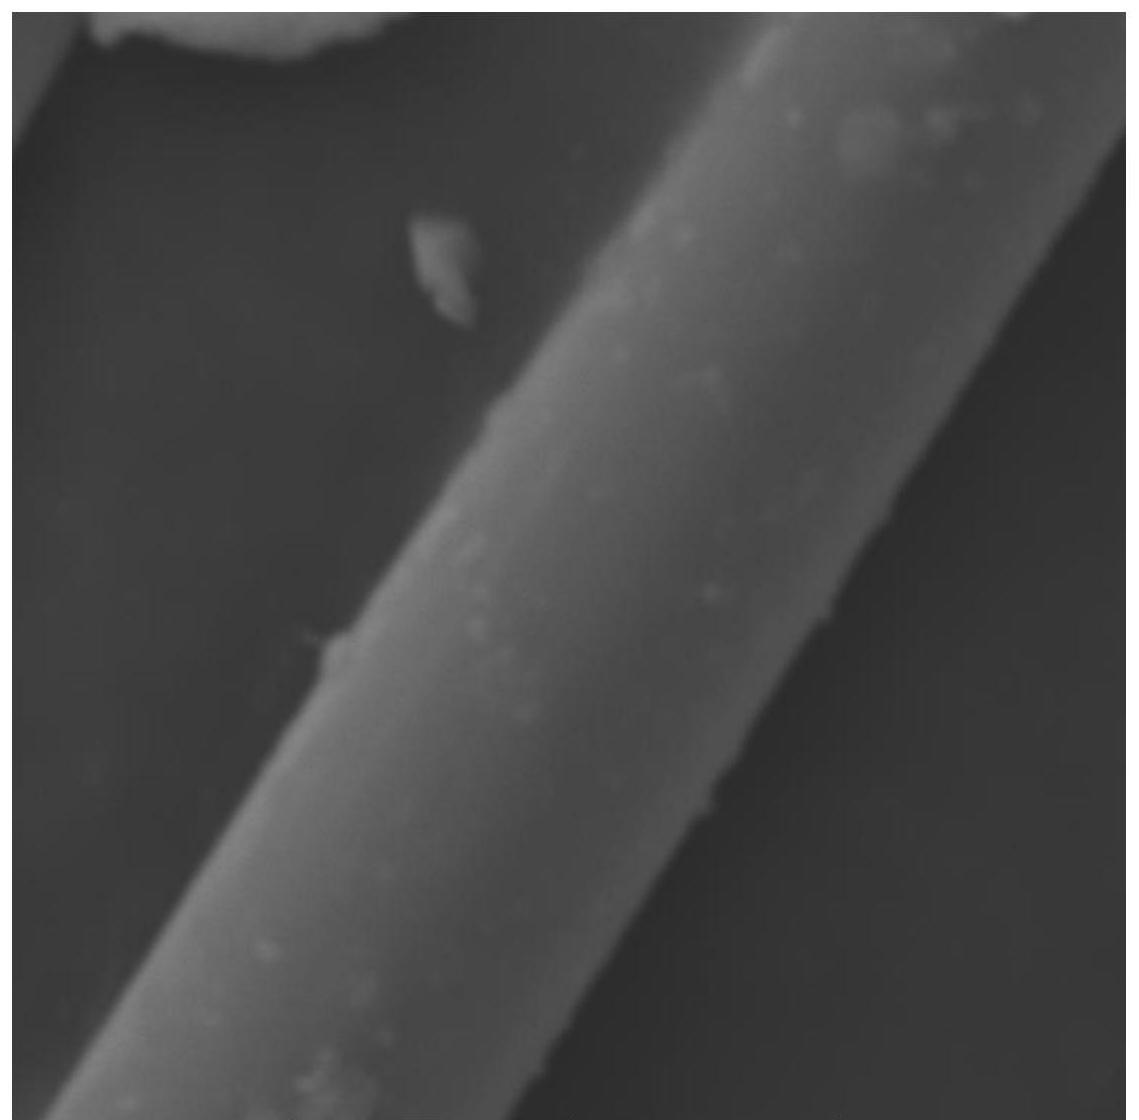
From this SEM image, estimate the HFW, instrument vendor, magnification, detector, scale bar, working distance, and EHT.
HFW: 41.5 µm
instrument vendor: Tescan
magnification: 5 K X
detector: SE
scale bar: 10000 nm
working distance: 24.11 mm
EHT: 20 kV